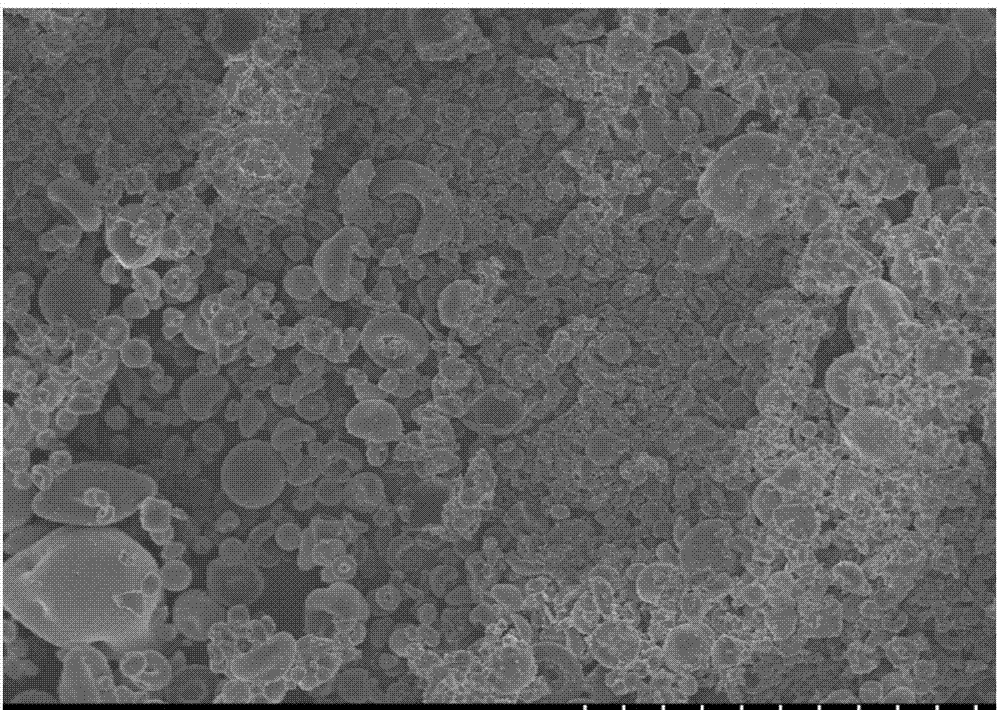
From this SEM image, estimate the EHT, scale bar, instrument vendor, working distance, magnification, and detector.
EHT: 3 kV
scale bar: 50000 nm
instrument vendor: Hitachi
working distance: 4.1 mm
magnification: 1 K X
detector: SE(U,LA0)